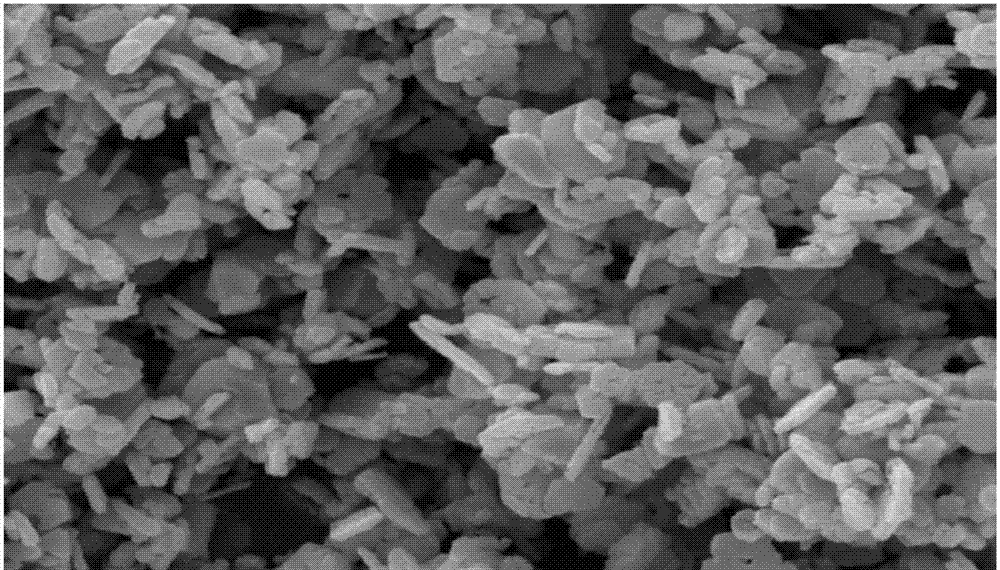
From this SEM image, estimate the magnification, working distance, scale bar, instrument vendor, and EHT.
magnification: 10 K X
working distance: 14.63 mm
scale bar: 2000 nm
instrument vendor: Tescan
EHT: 15 kV